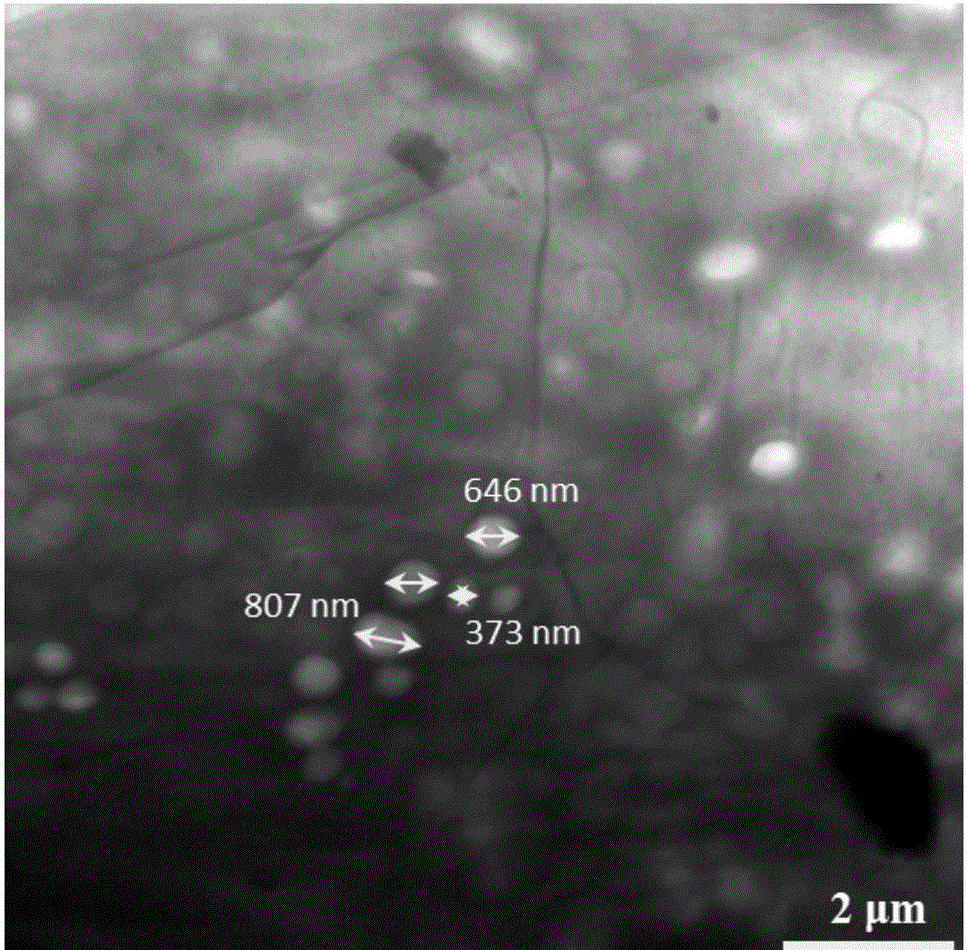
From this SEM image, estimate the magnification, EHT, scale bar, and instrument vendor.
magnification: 2 K X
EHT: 100 kV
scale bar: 2000 nm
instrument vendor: Hitachi